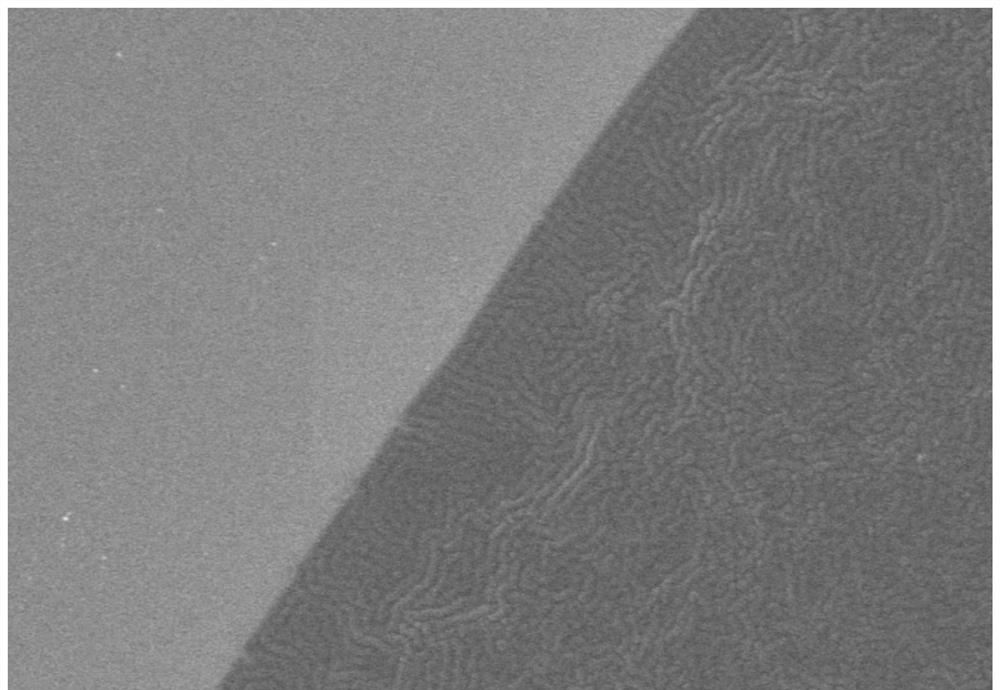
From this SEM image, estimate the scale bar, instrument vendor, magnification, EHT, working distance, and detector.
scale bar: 50000 nm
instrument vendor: Hitachi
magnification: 1 K X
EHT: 5 kV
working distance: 3.6 mm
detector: LM(UL)